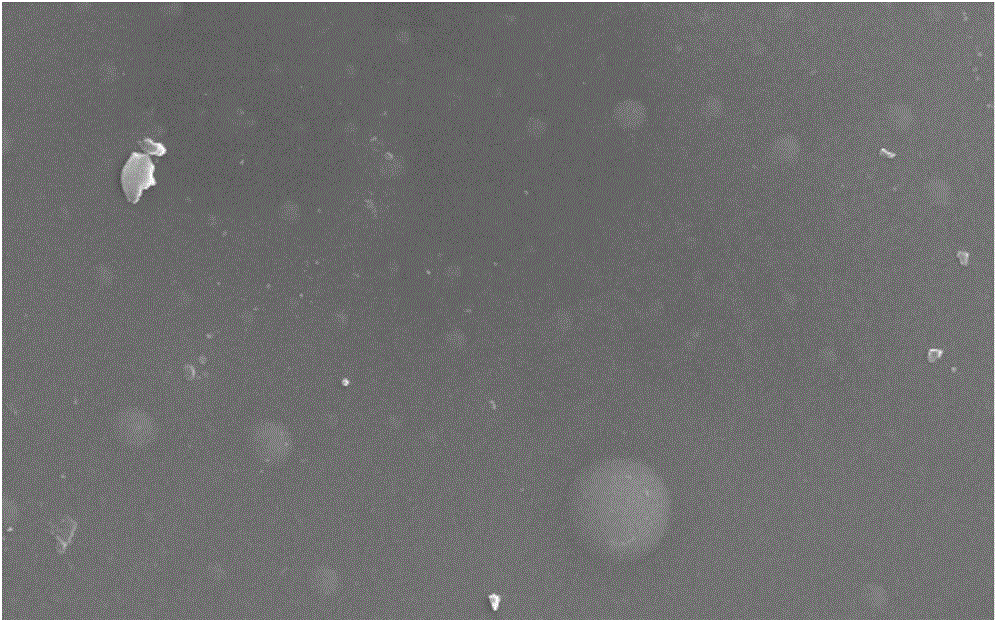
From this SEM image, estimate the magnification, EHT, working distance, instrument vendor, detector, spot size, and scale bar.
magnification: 2 K X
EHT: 25 kV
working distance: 7.1 mm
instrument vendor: FEI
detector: SE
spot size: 4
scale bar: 20000 nm